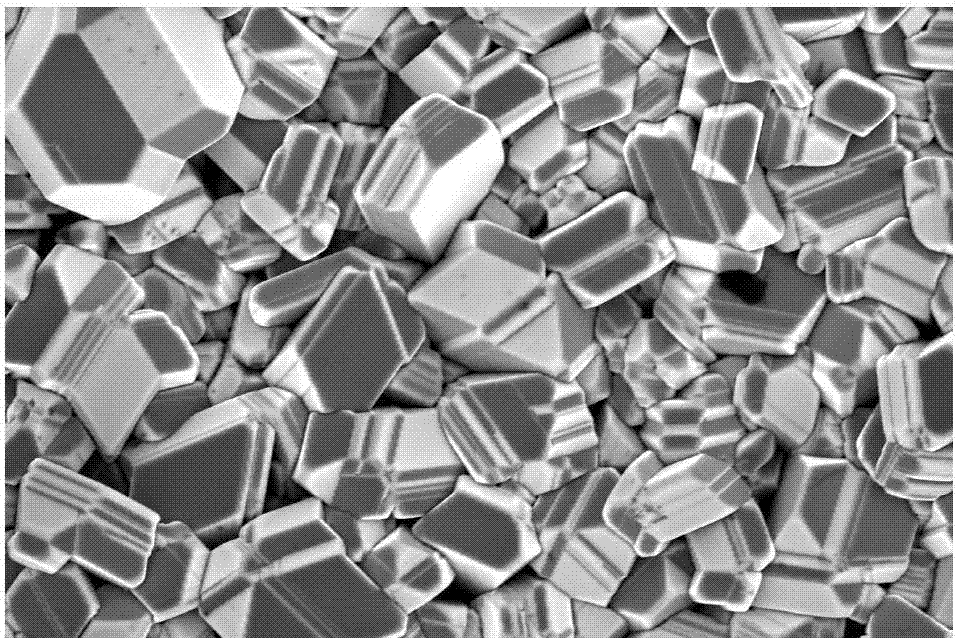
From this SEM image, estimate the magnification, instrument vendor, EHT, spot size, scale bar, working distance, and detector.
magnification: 25 K X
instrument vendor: FEI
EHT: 5 kV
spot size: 3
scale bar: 5000 nm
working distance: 5.3 mm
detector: TLD